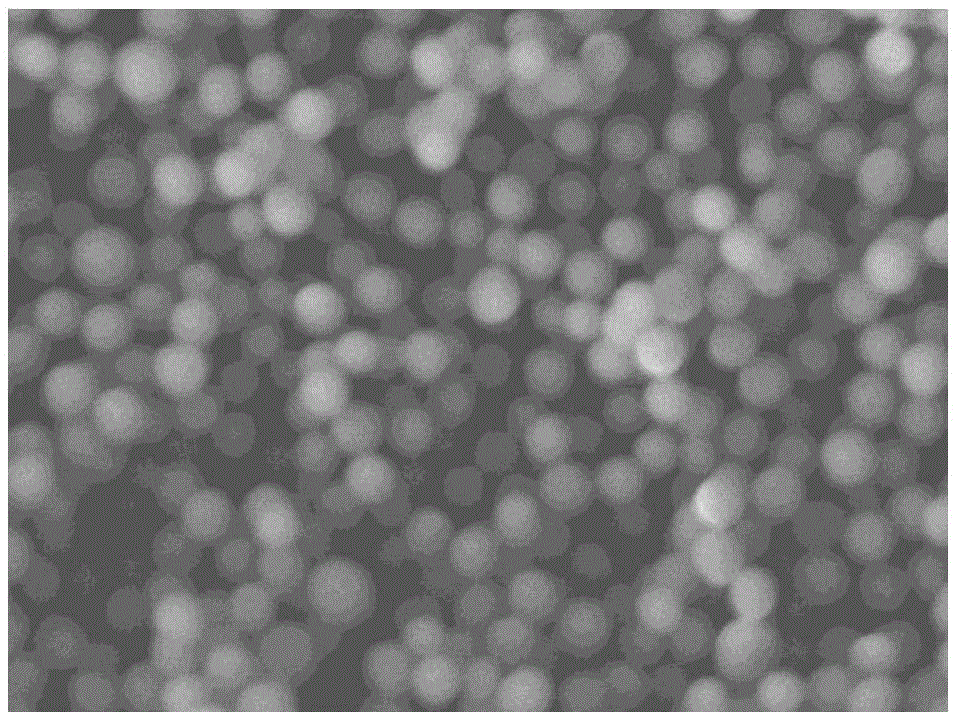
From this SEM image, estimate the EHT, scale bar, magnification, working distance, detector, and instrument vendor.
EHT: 3 kV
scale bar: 100 nm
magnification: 50 K X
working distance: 7.8 mm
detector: LEI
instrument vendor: JEOL